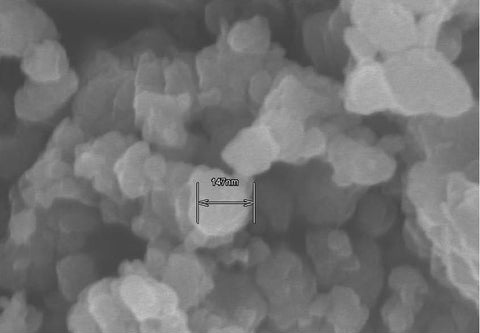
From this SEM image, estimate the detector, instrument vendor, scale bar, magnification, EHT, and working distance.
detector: SE(M)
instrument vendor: Hitachi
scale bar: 500 nm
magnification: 100 K X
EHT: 5 kV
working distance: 9.5 mm